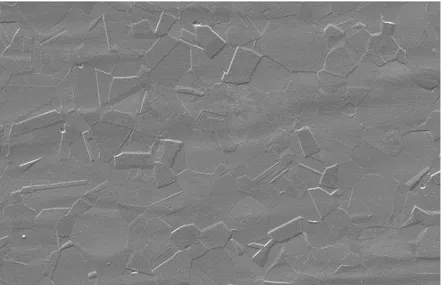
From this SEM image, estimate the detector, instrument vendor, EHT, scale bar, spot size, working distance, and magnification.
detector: SE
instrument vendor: FEI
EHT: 20 kV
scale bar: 100000 nm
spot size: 6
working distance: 11.5 mm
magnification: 0.5 K X